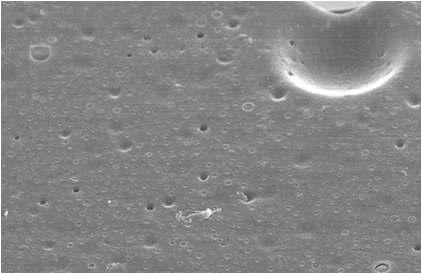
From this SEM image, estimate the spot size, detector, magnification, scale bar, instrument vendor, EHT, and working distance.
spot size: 3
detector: TLD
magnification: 8 K X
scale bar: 10000 nm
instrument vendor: FEI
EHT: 10 kV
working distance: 5.1 mm